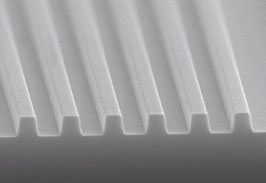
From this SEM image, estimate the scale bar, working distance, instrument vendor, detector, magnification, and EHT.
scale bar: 1000 nm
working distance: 9.3 mm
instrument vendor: Hitachi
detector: S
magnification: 10 K X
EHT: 20 kV